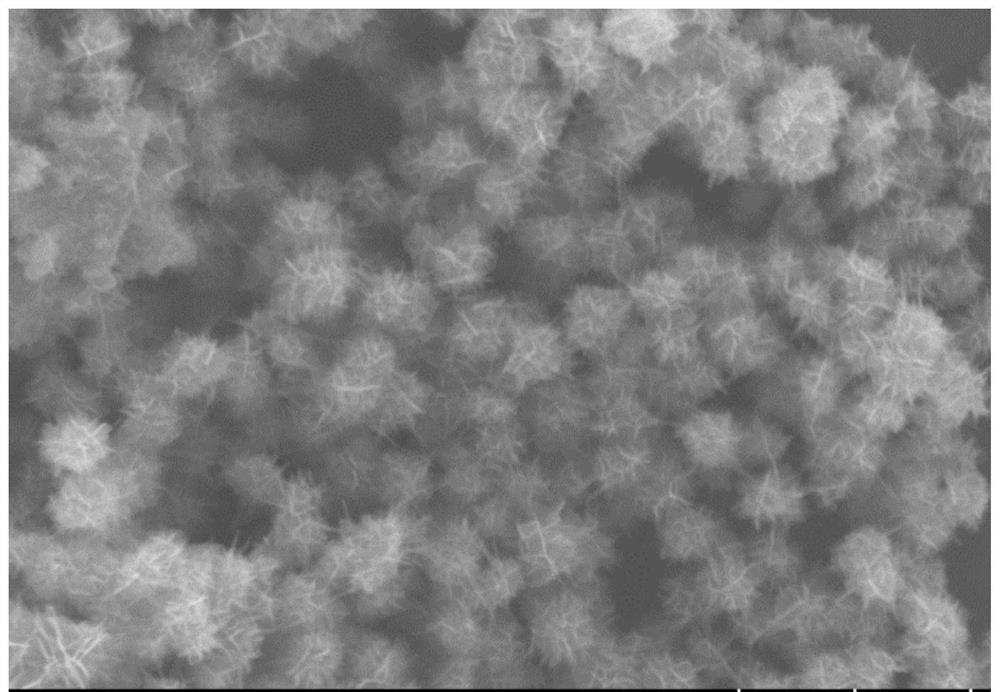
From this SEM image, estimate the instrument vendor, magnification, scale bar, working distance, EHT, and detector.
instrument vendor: Hitachi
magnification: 30 K X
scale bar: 1000 nm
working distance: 9.7 mm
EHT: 5 kV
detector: SE(UL)